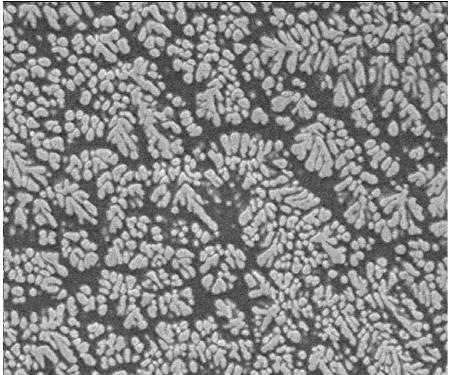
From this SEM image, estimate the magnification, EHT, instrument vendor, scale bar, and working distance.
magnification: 5 K X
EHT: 5 kV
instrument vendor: JEOL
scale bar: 1000 nm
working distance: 13 mm